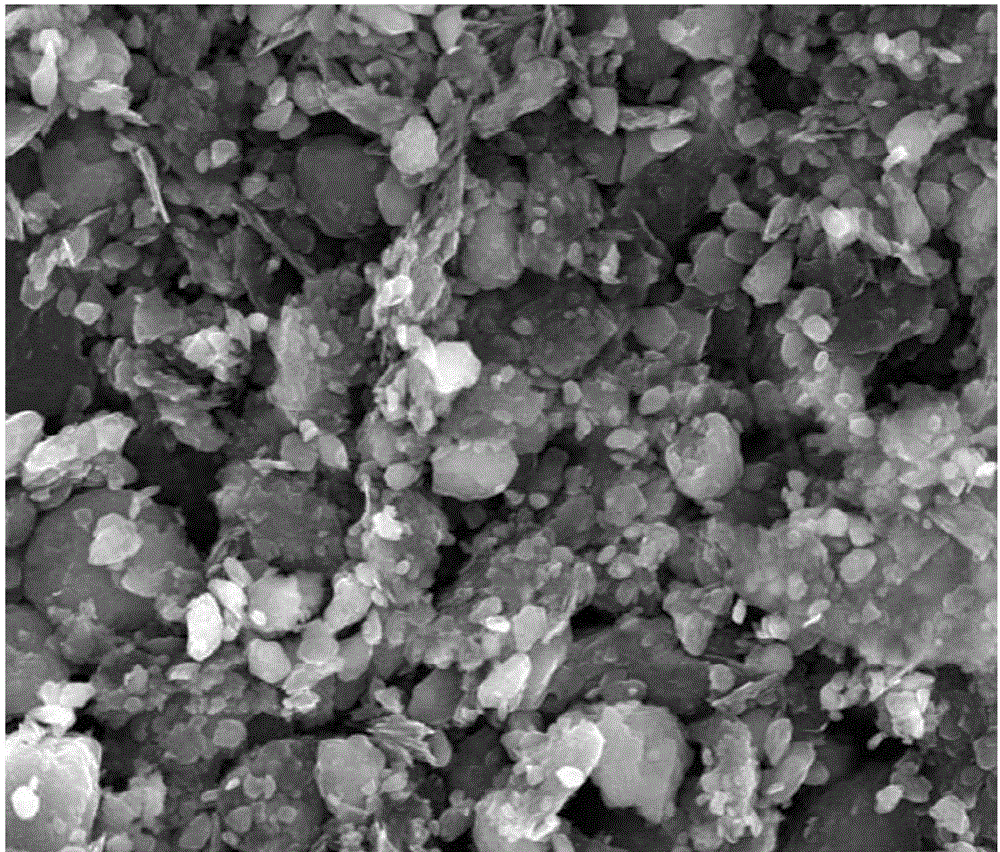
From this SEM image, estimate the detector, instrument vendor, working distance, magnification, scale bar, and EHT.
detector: TLD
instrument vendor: FEI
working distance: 5.1 mm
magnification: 40 K X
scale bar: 2000 nm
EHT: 10 kV